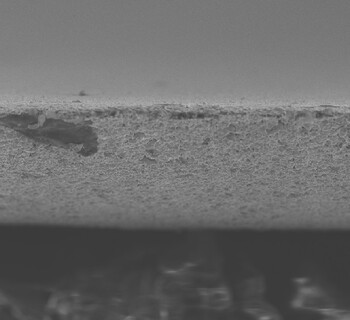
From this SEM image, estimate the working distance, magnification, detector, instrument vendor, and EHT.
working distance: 9.9 mm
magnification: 1 K X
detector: SE(UL)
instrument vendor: Hitachi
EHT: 10 kV